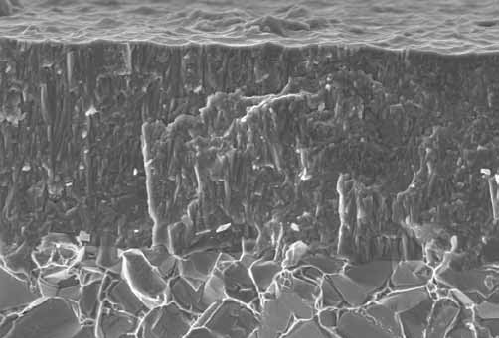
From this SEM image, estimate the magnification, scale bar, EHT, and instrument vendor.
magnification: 10 K X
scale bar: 2000 nm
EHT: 4 kV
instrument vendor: Hitachi